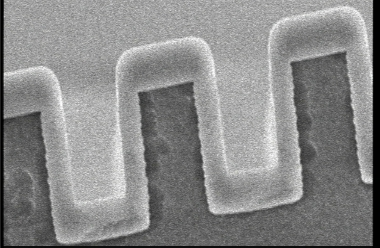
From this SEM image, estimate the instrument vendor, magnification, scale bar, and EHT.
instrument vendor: JEOL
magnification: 9.5 K X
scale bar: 2000 nm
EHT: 10 kV